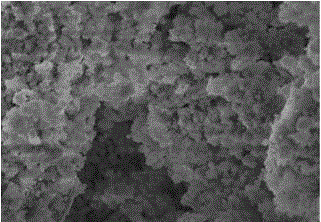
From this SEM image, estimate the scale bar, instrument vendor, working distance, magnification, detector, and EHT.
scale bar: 2000 nm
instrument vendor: Hitachi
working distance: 7.6 mm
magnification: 20 K X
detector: SE(M)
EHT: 5 kV